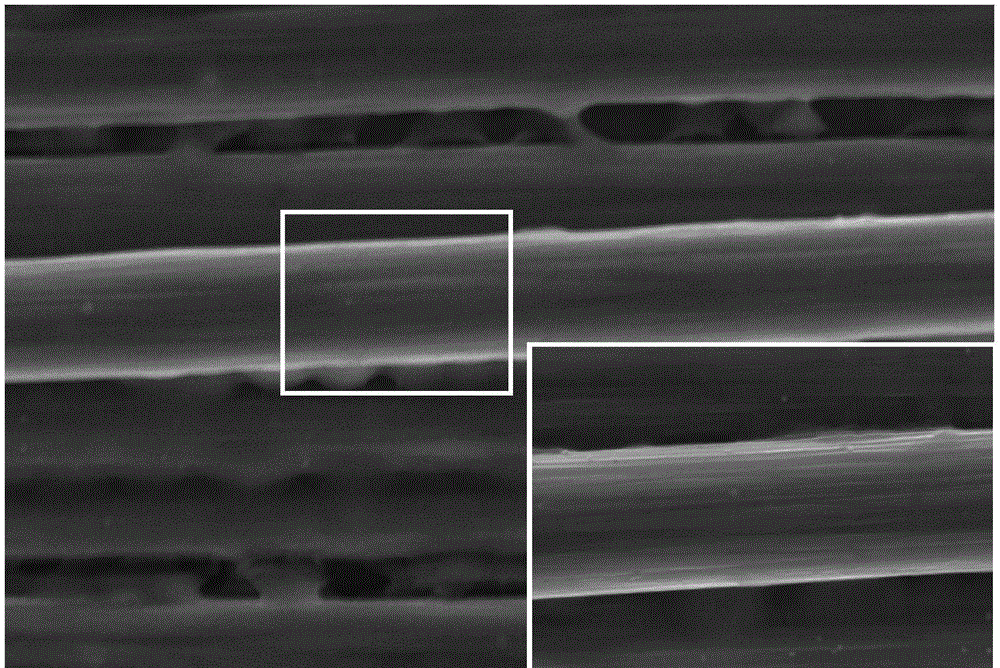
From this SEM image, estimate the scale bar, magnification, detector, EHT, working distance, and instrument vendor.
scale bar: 20000 nm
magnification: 2 K X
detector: SE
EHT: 15 kV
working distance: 11.9 mm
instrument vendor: Hitachi